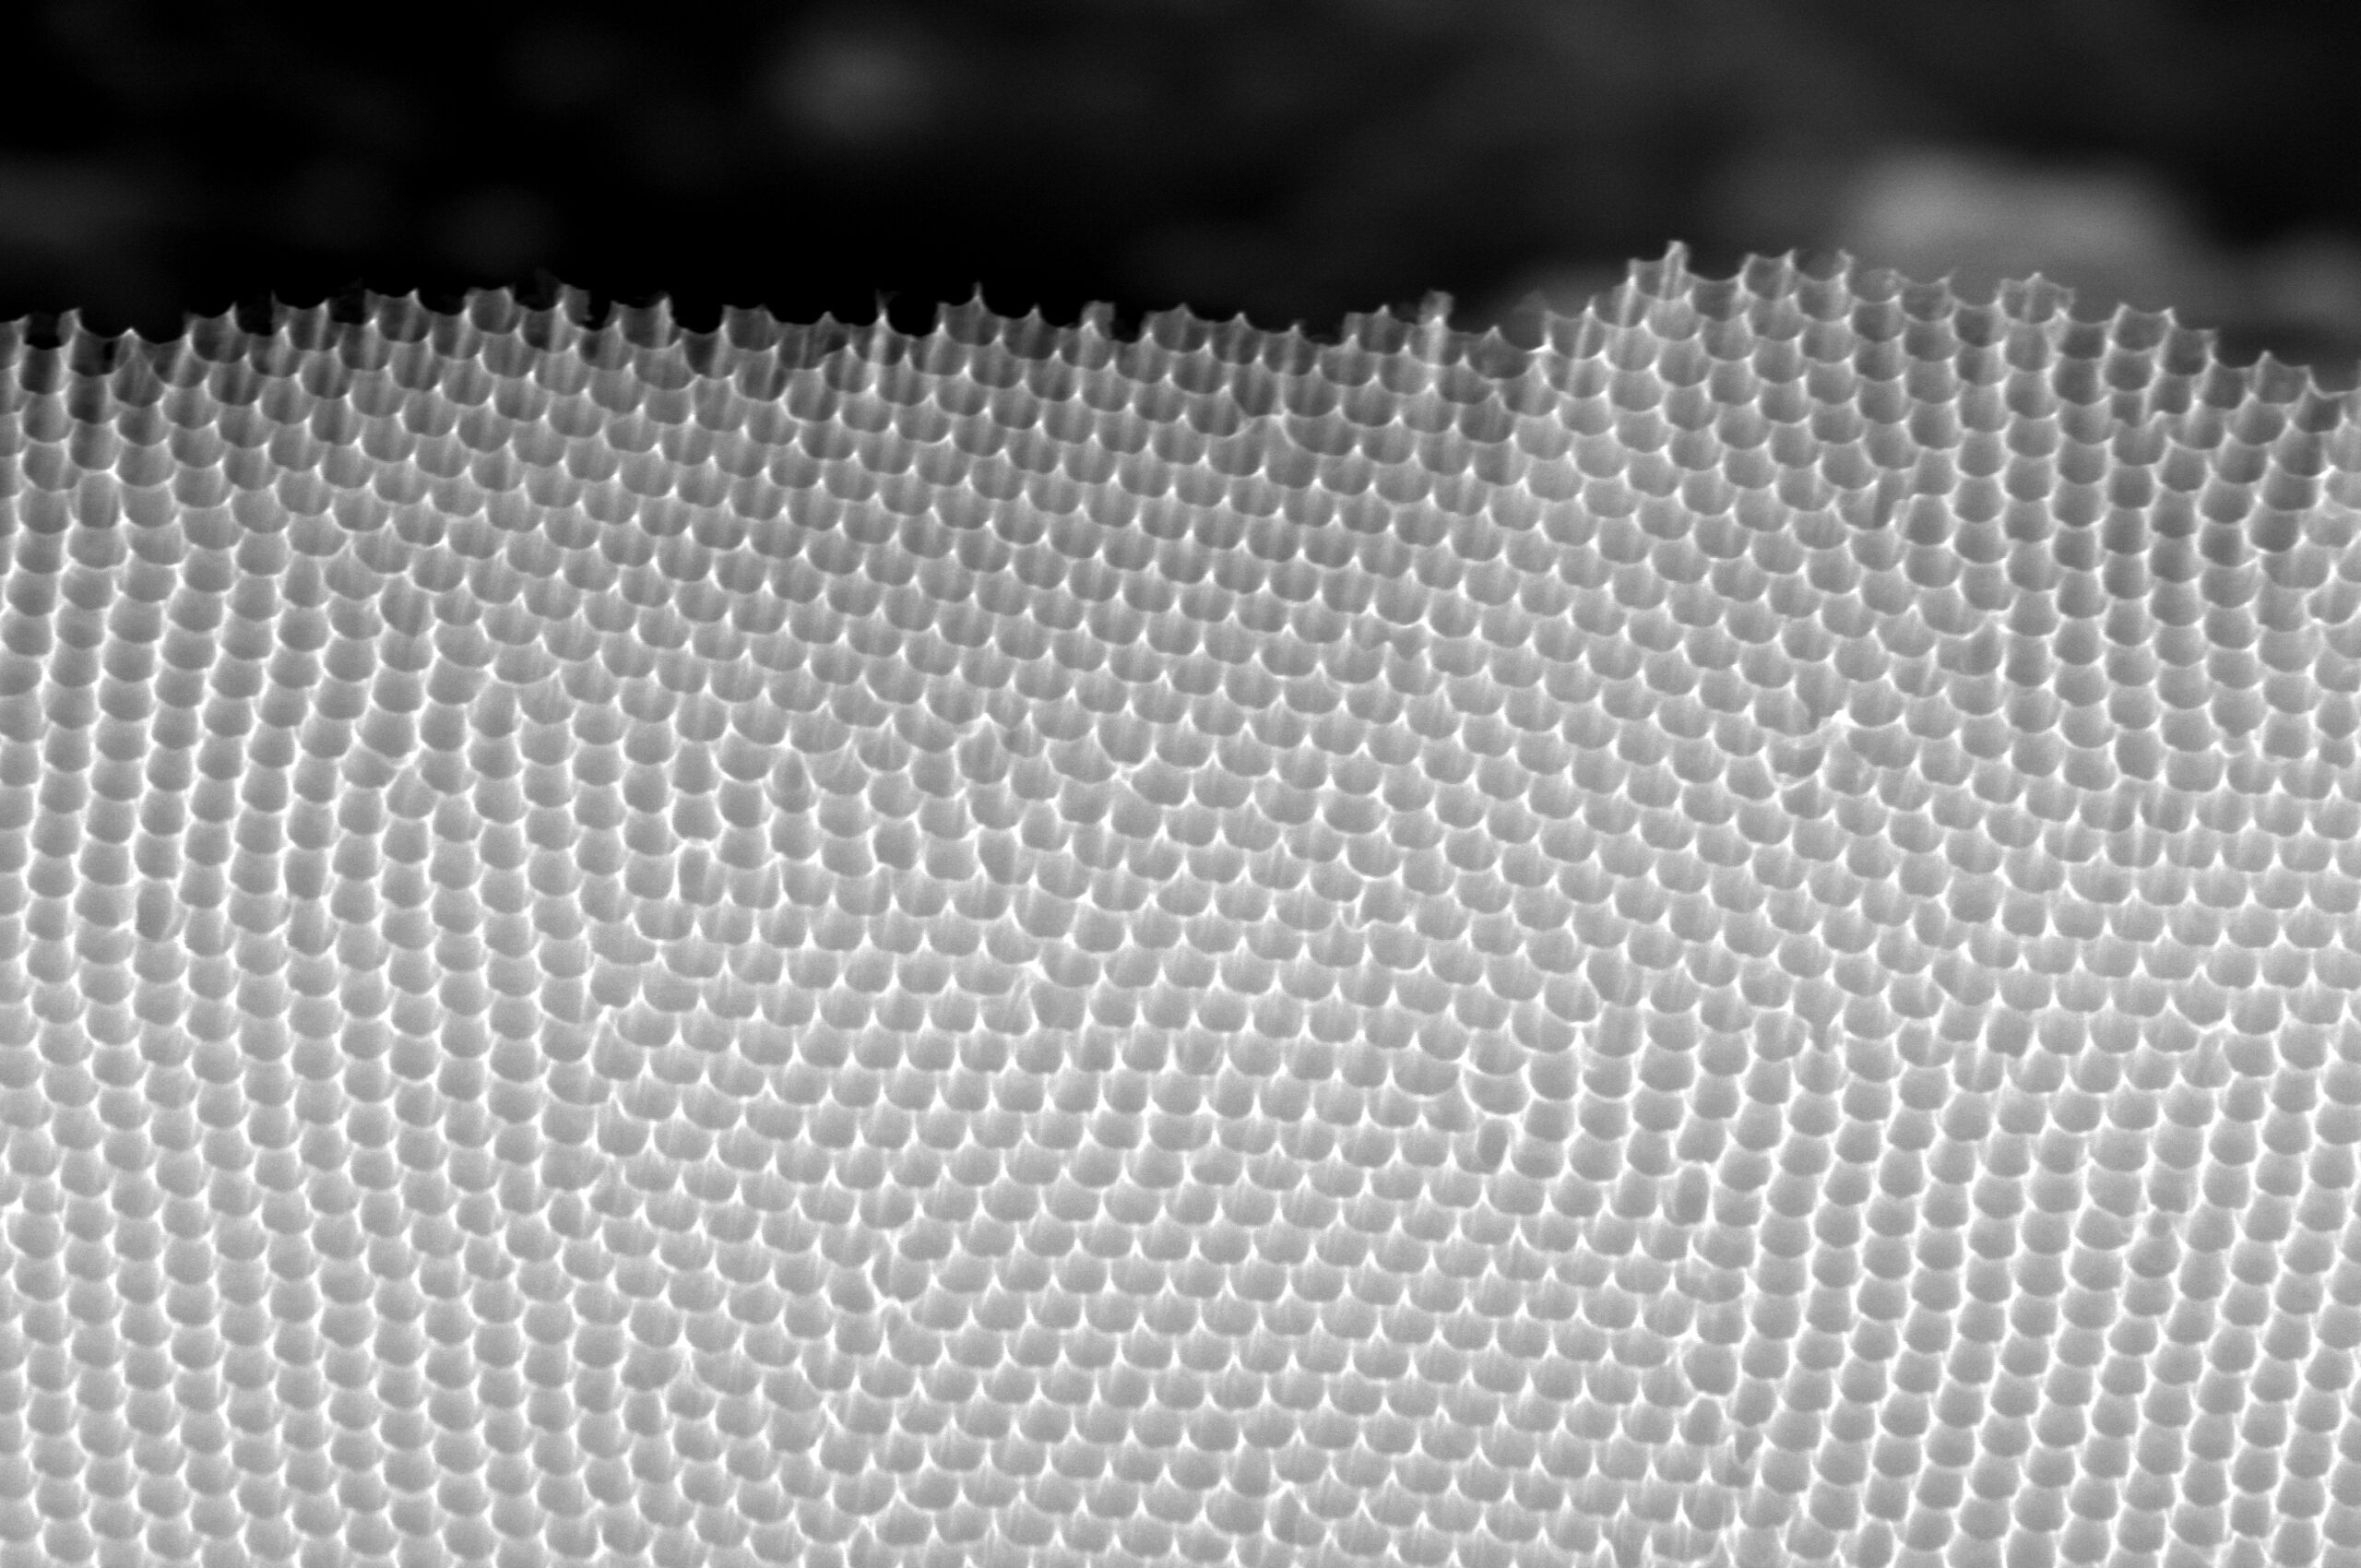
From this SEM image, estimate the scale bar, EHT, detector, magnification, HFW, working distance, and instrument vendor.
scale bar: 1000 nm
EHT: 20 kV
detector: ETD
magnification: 100 K X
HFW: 4.14 µm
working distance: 6.3 mm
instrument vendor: FEI